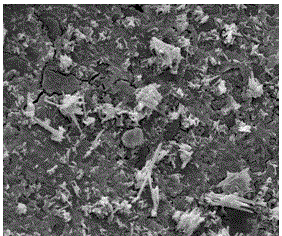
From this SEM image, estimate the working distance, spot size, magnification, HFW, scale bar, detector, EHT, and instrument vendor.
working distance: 6.3 mm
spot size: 2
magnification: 5 K X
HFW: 29.8 µm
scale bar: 5000 nm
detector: ETD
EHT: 10 kV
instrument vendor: FEI